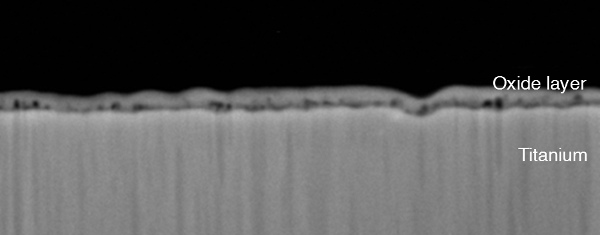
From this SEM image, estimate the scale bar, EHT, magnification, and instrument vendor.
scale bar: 1000 nm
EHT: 10 kV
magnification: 10 K X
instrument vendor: JEOL